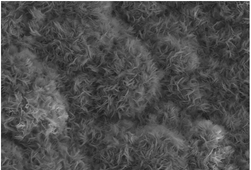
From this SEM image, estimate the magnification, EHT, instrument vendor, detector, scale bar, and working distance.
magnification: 13 K X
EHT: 5 kV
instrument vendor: Hitachi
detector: SE(M)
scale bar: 4000 nm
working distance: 14.9 mm